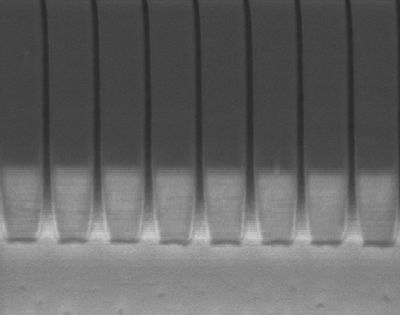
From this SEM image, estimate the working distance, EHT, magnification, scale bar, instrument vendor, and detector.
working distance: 3.9 mm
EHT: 0.7 kV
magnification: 50 K X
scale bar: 100 nm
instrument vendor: Zeiss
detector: InLens